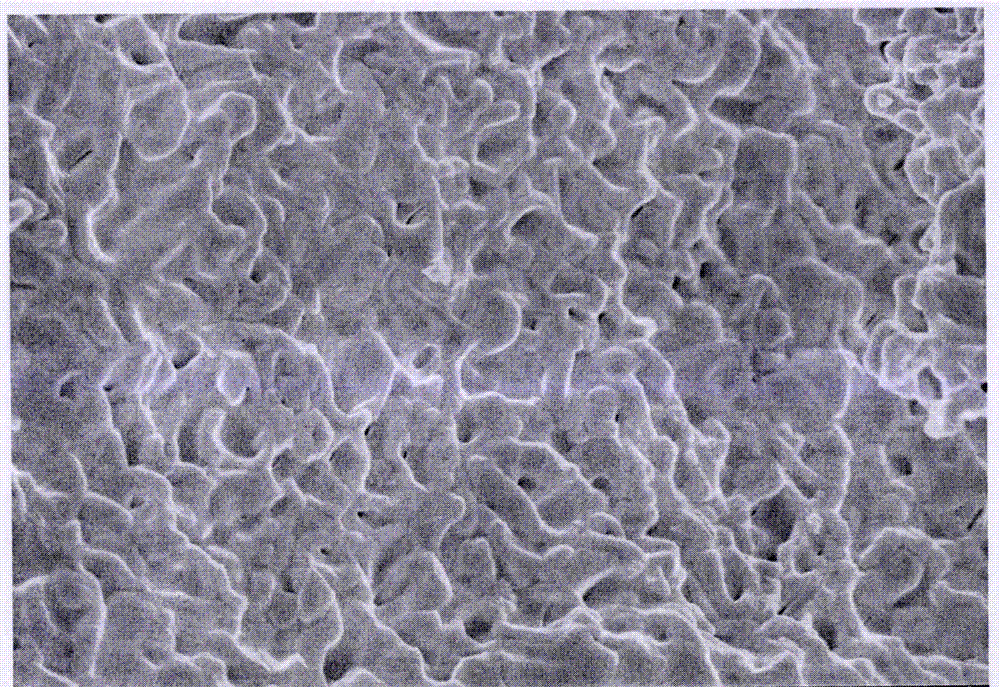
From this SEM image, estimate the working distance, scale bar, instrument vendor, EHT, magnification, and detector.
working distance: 8.5 mm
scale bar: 3000 nm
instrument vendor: Hitachi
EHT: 5 kV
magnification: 15 K X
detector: SE(U)